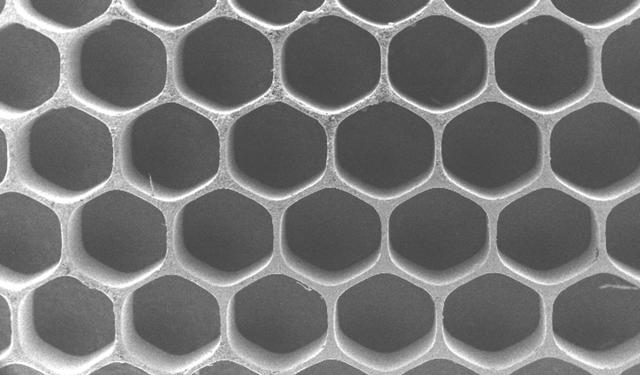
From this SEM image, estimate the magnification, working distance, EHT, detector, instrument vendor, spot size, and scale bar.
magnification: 0.034 K X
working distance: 6.3 mm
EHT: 5 kV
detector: SE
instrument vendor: FEI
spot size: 4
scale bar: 1000000 nm